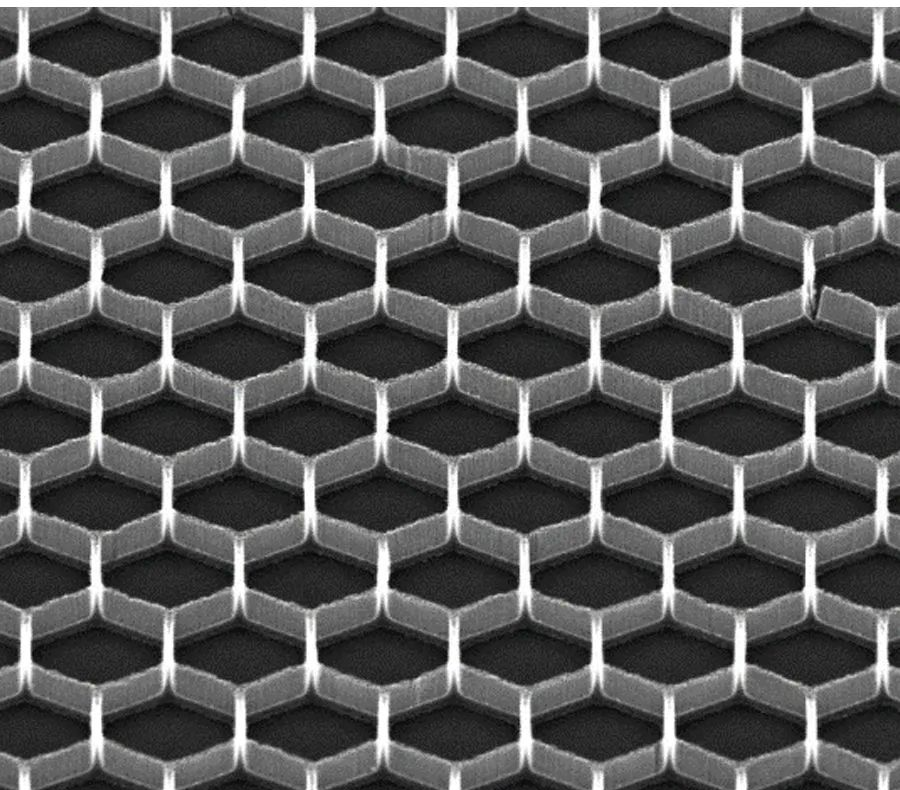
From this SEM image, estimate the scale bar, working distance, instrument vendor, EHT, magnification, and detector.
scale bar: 20000 nm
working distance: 13.5 mm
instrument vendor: FEI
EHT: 20 kV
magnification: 5 K X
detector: SE